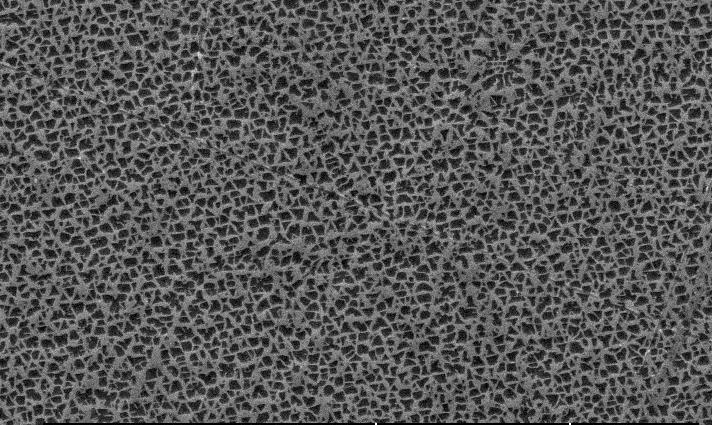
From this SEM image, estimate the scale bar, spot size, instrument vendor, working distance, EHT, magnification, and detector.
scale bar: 10000 nm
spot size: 4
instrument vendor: FEI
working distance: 8.3 mm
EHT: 20 kV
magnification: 2.5 K X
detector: SE